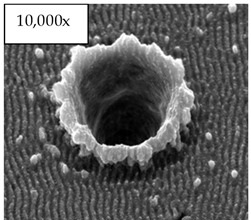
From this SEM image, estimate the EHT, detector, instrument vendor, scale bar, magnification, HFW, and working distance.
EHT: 30 kV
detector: ETD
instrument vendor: FEI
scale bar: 10000 nm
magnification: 10 K X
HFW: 29.8 µm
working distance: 9.3 mm